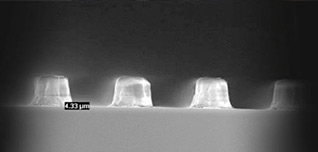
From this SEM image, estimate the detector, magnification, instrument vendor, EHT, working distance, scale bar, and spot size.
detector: TLD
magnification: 8 K X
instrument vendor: FEI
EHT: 10 kV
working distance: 3.4 mm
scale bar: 10000 nm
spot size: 3